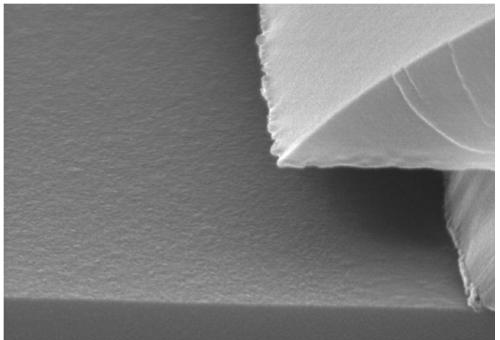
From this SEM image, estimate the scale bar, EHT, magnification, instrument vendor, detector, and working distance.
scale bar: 500 nm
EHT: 30 kV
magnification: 60 K X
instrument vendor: Hitachi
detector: SE(M)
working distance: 10.2 mm